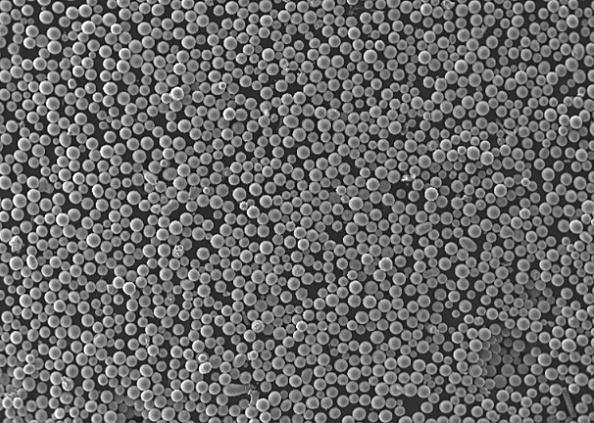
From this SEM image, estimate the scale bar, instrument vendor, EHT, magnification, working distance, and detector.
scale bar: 500000 nm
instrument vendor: Tescan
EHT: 30 kV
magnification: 0.1 K X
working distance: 5.47 mm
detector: SE